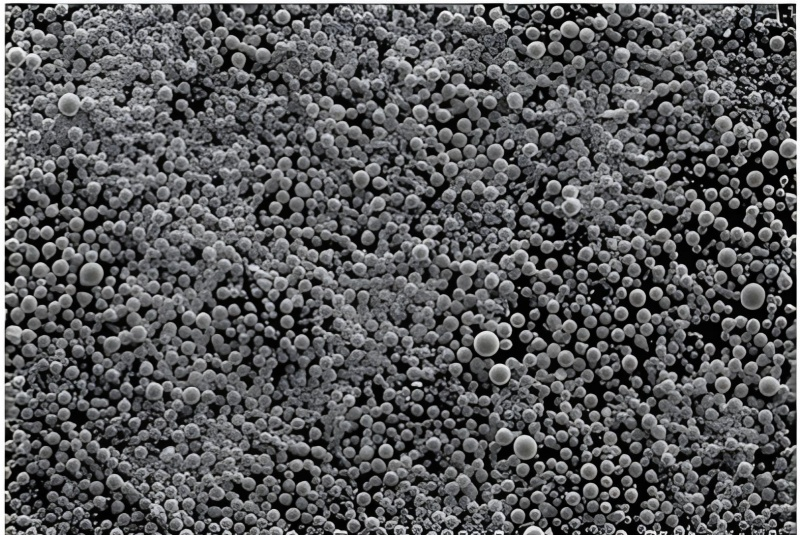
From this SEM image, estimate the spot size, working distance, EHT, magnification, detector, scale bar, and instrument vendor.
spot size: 12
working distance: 8.7 mm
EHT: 15 kV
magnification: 0.2 K X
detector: SE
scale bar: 100000 nm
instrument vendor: JEOL